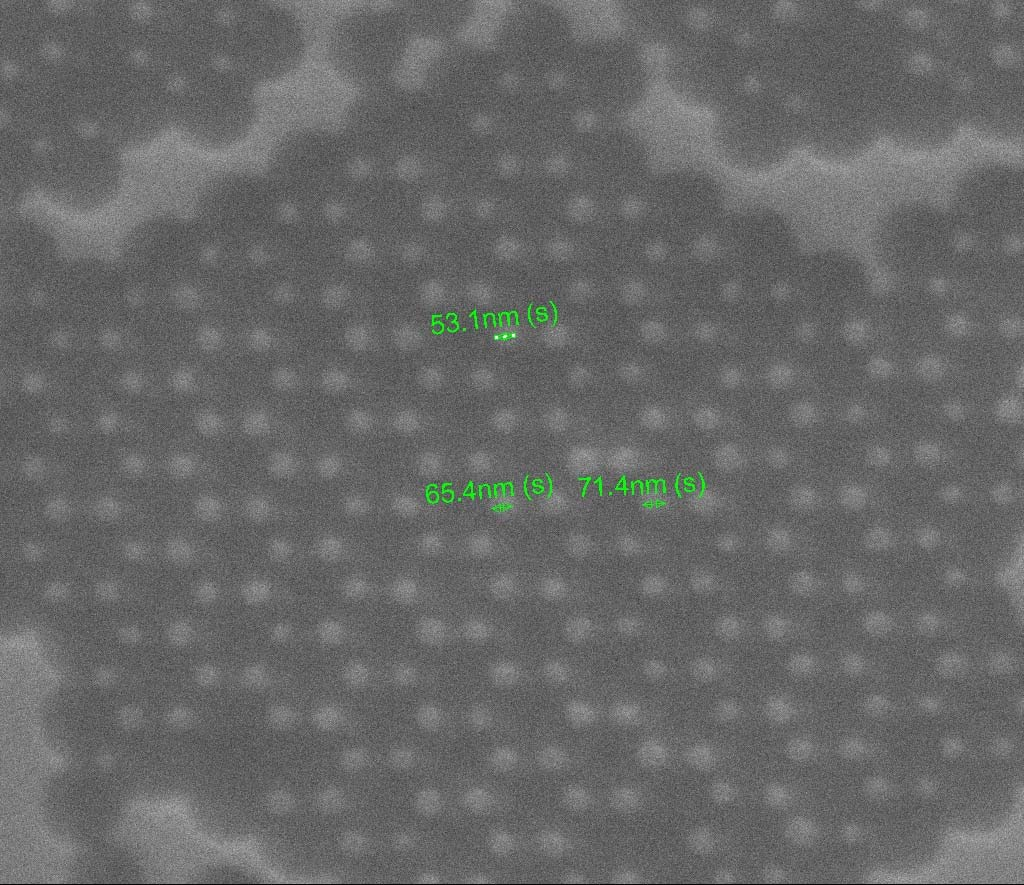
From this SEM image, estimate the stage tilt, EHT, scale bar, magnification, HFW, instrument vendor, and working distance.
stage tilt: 1°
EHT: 25 kV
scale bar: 1000 nm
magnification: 85.174 K X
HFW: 3.17 µm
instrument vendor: FEI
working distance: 11.8 mm